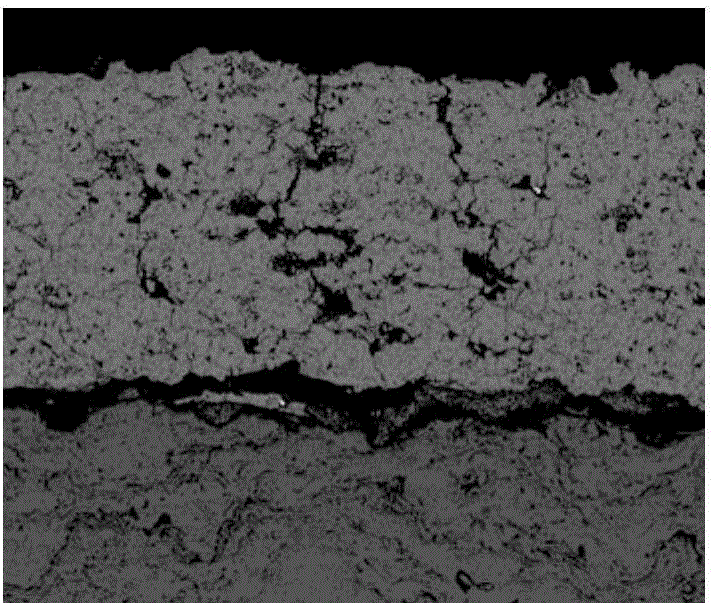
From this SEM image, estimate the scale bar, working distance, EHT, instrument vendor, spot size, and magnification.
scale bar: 200000 nm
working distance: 13.3 mm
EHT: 20 kV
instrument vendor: FEI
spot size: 4.5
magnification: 0.5 K X